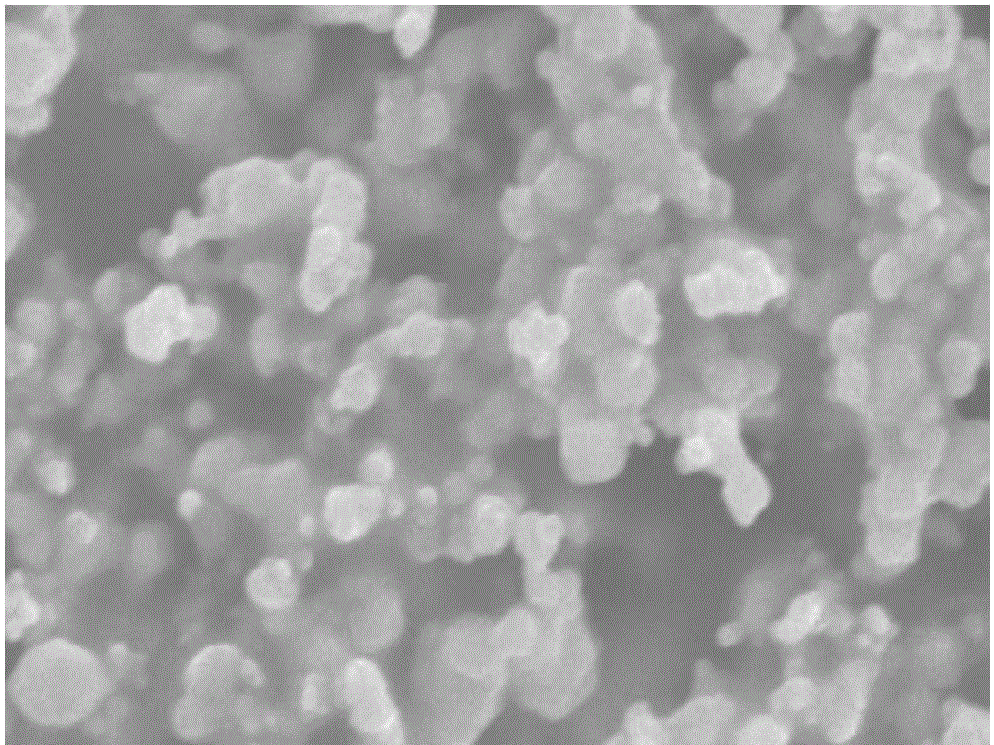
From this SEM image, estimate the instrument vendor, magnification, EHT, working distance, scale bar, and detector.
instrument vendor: Hitachi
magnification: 19.1 K X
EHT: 15 kV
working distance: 9.6 mm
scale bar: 3000 nm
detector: SE